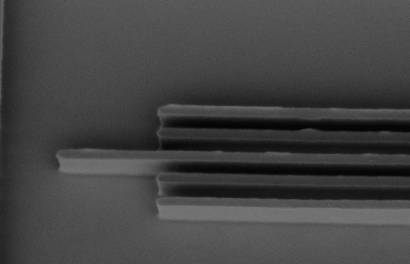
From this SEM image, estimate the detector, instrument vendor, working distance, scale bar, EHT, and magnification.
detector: InLens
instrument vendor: Zeiss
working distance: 5.8 mm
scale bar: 1000 nm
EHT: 1.4 kV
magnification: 55.66 K X